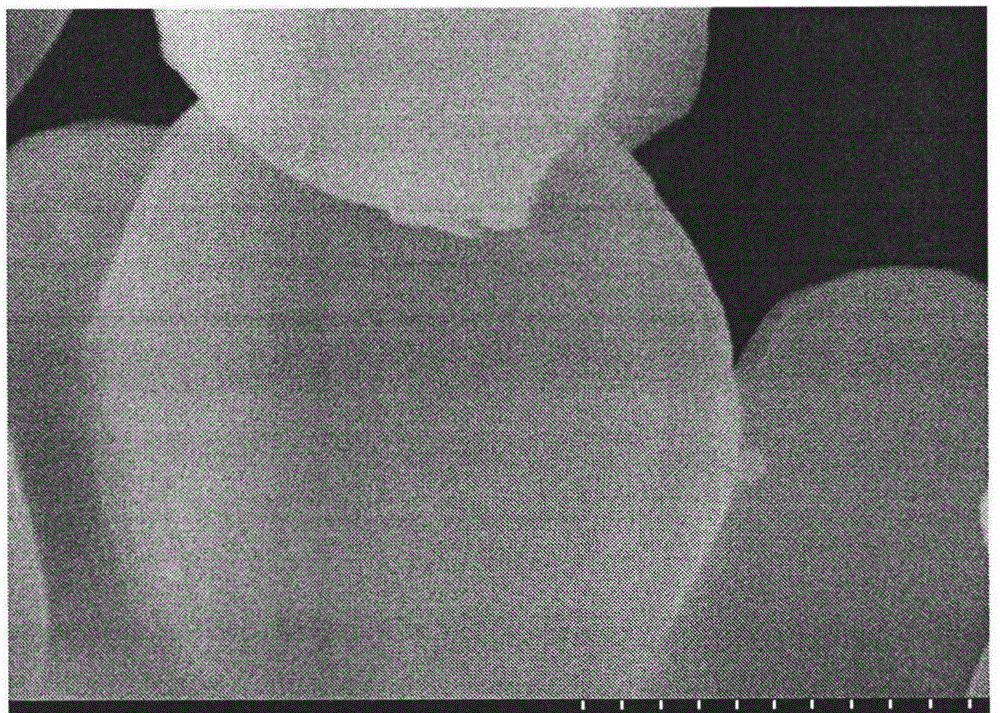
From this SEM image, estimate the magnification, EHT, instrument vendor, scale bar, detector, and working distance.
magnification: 50 K X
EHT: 5 kV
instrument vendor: Hitachi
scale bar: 1000 nm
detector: SE(M)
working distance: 10.5 mm